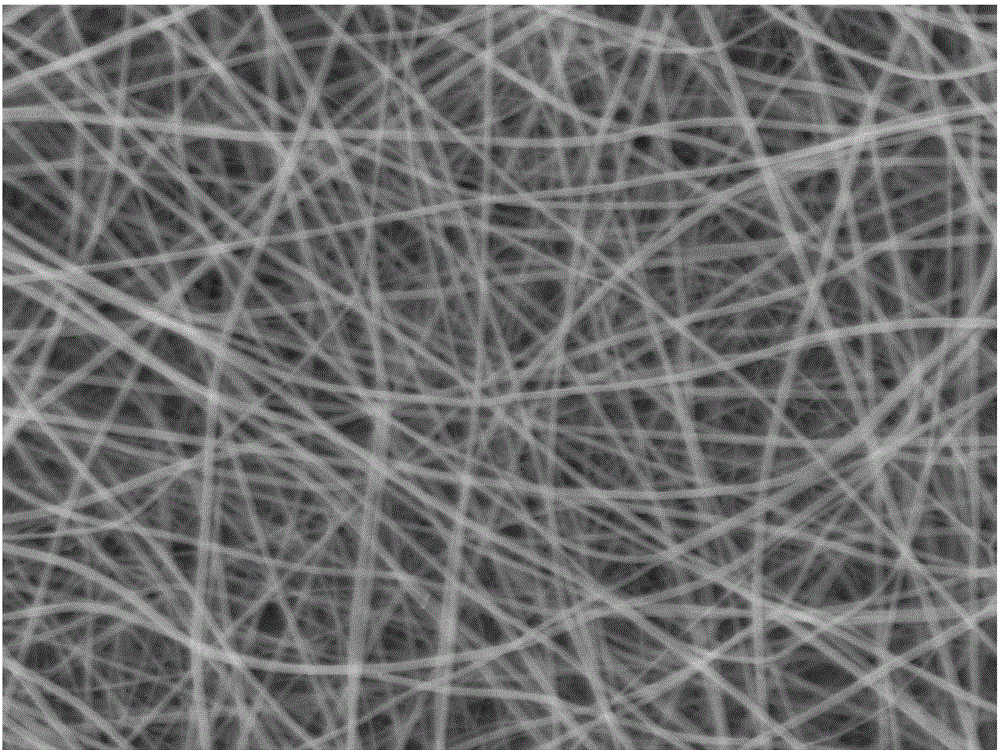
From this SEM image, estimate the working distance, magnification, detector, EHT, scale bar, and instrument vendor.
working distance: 15.1 mm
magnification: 5 K X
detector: SEI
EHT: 20 kV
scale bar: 1000 nm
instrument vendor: JEOL